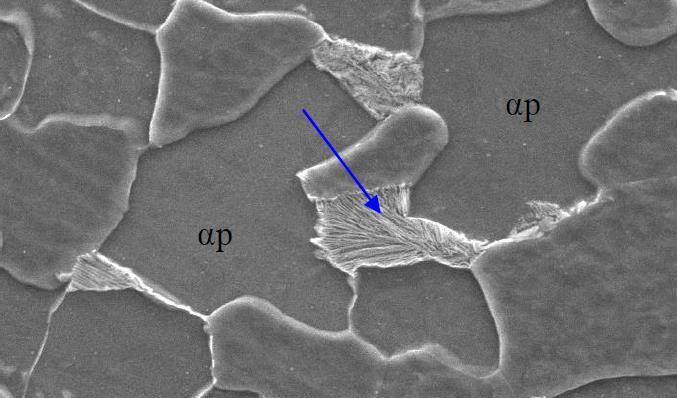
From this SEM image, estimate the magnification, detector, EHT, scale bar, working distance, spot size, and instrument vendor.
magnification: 6.5 K X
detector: SE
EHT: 20 kV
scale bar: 10000 nm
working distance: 10.1 mm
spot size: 4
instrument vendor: FEI